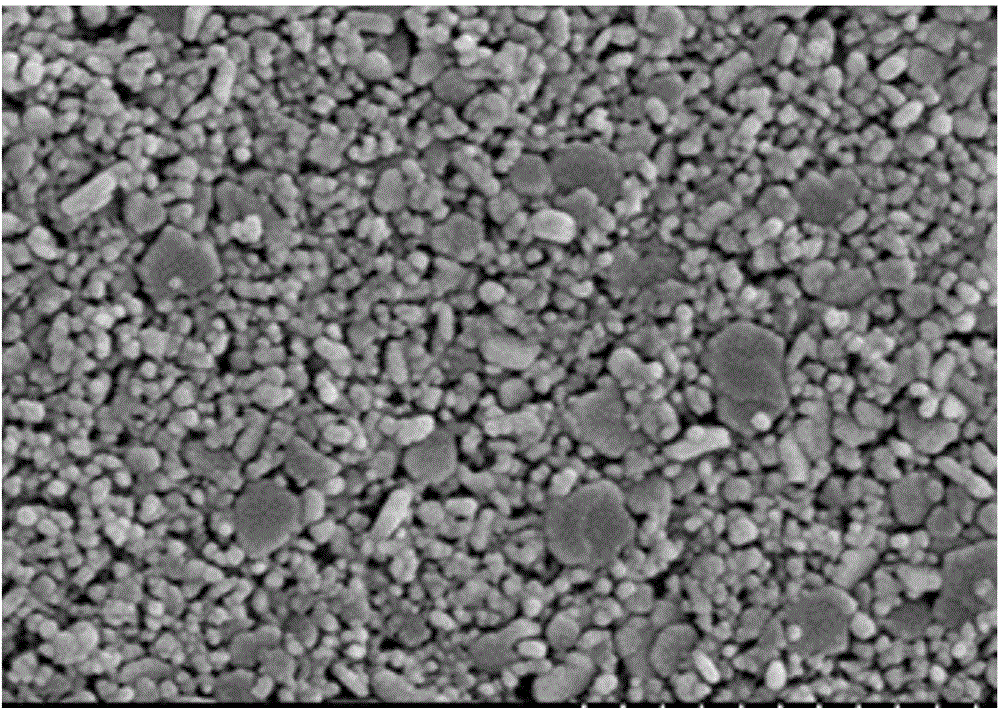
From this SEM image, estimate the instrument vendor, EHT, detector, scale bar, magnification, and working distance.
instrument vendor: Hitachi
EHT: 5 kV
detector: SE(M)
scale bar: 1000 nm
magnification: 50 K X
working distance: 9.7 mm